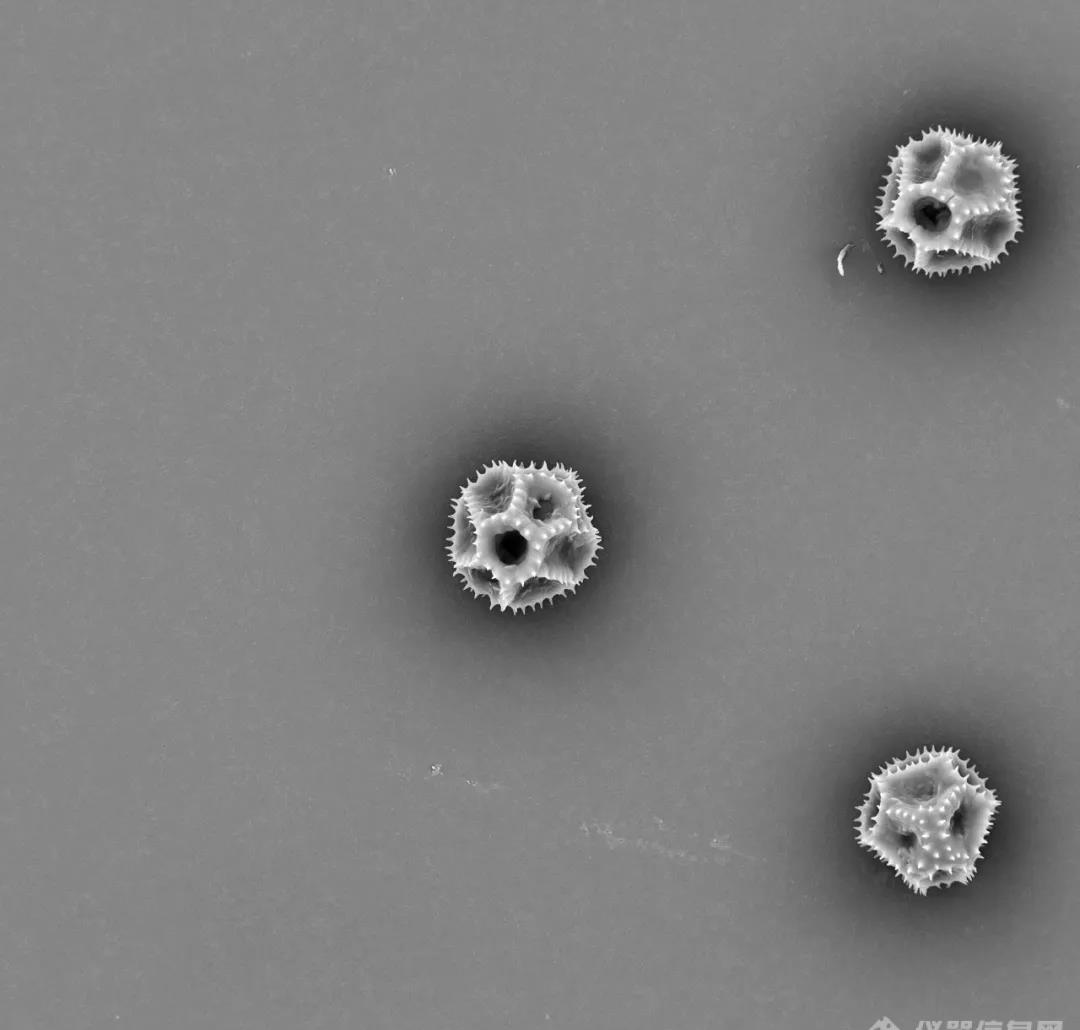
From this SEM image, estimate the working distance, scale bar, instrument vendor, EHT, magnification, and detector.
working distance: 5.5 mm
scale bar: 50000 nm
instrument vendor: other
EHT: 15 kV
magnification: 1 K X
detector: BSE